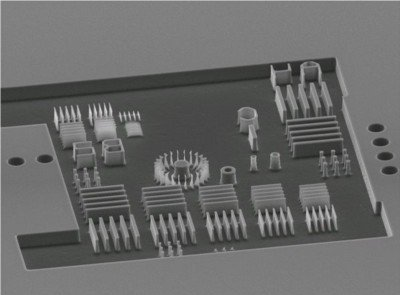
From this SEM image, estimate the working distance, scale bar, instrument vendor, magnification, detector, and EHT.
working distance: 27 mm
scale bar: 10000 nm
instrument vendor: JEOL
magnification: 0.45 K X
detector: SEI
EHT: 15 kV